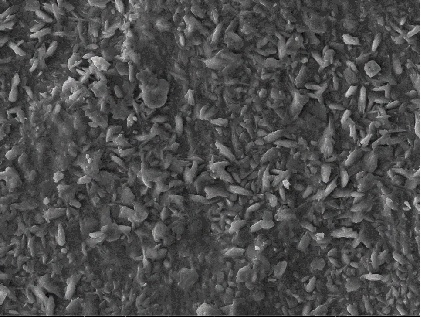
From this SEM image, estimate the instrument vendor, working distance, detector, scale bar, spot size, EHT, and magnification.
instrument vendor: other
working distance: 3.95 mm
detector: SEI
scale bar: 4000 nm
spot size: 1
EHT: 20 kV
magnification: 3 K X